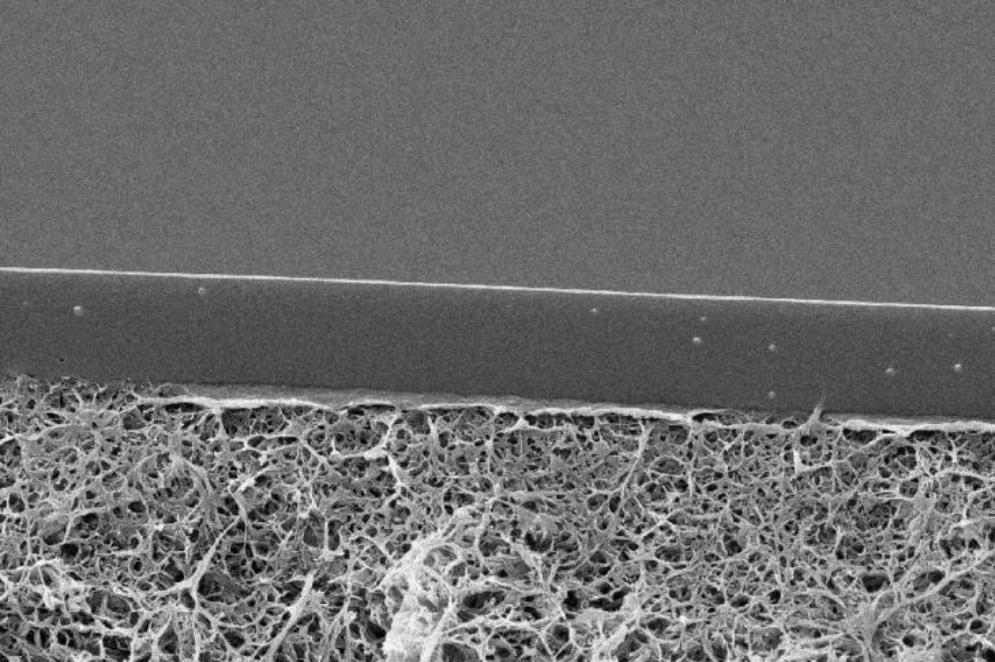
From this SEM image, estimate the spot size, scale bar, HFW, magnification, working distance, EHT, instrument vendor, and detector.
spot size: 8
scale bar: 5000 nm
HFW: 25.9 µm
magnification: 8 K X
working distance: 10.7 mm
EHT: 8 kV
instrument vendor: Thermo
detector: ETD